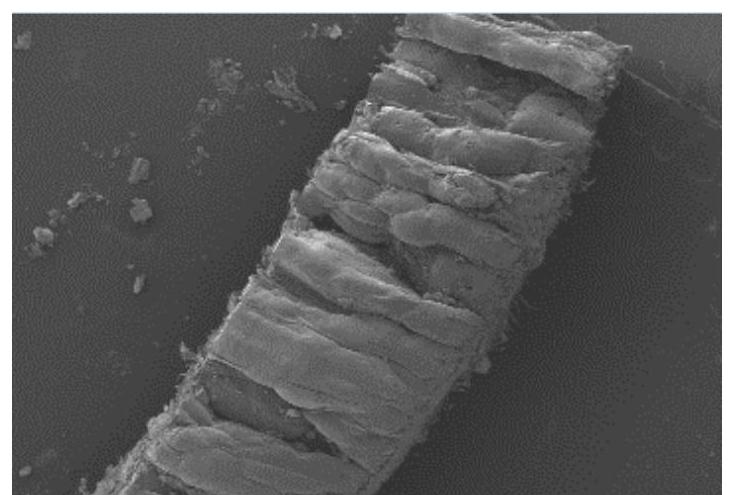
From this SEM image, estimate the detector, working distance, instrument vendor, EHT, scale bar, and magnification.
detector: LM(L)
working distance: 9.5 mm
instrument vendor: Hitachi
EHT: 3 kV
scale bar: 1000000 nm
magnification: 0.03 K X